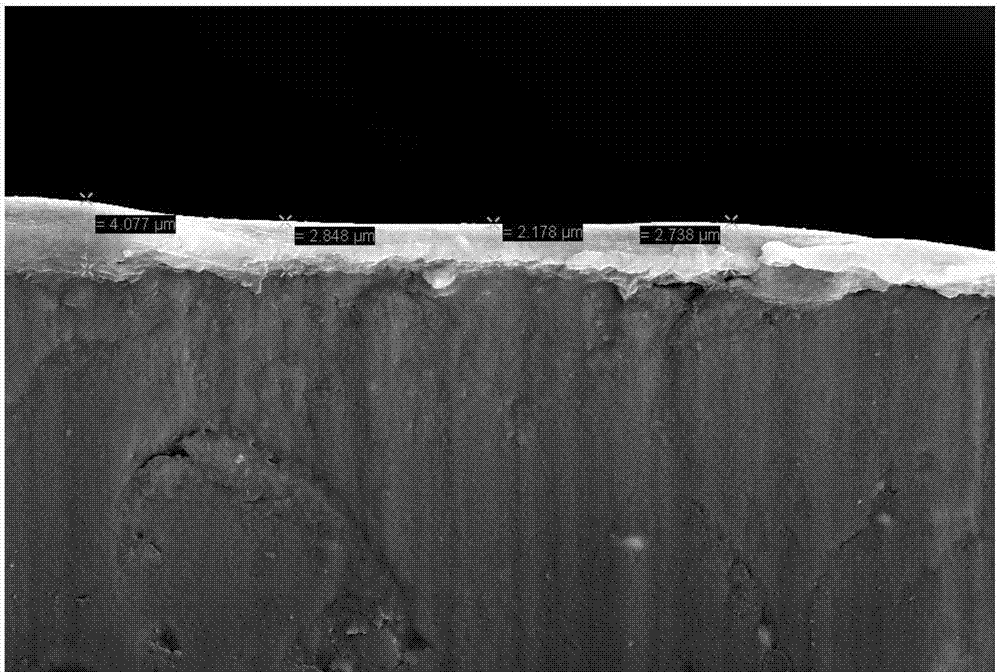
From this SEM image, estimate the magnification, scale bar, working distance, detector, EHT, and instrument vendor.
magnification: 2 K X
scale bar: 2000 nm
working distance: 7.7 mm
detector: SE2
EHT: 15 kV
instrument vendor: Zeiss